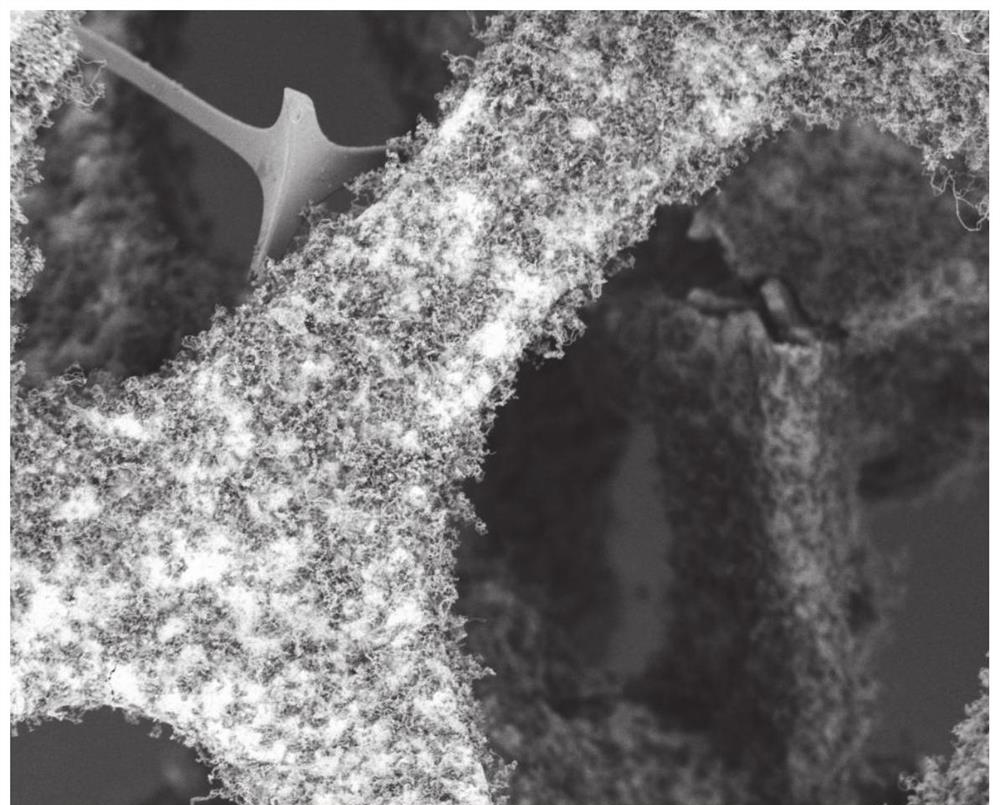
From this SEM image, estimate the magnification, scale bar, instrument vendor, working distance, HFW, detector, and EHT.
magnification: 1.3 K X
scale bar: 100000 nm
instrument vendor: Thermo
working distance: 6.4 mm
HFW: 319 µm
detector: T1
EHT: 2 kV